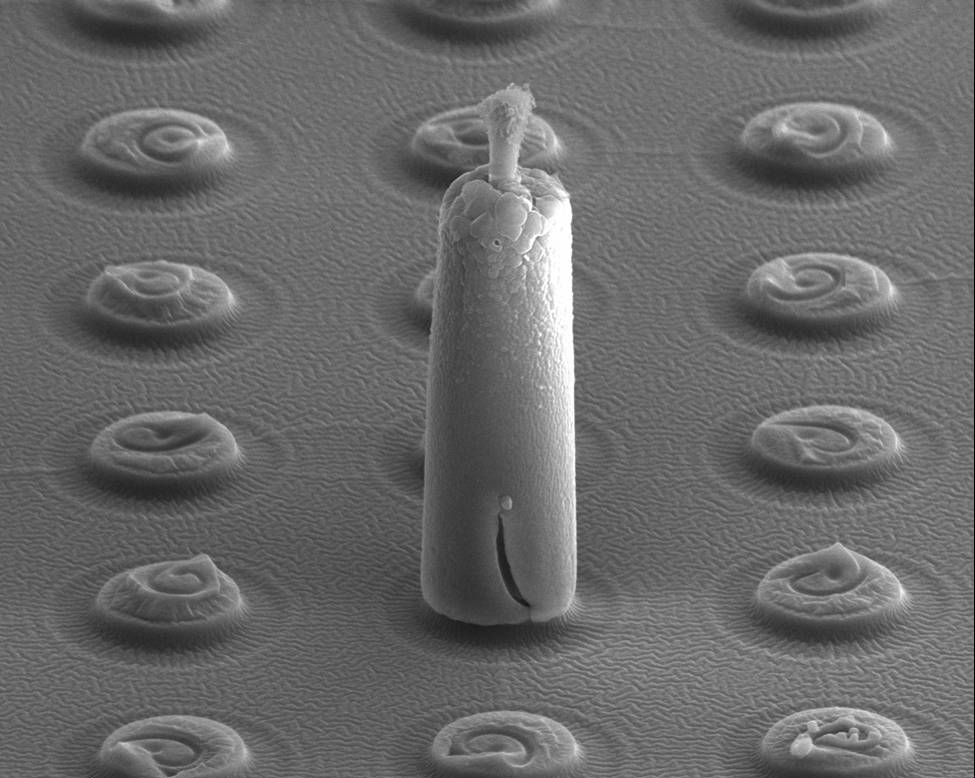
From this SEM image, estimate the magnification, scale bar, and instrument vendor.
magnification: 5 K X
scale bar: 50000 nm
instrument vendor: FEI